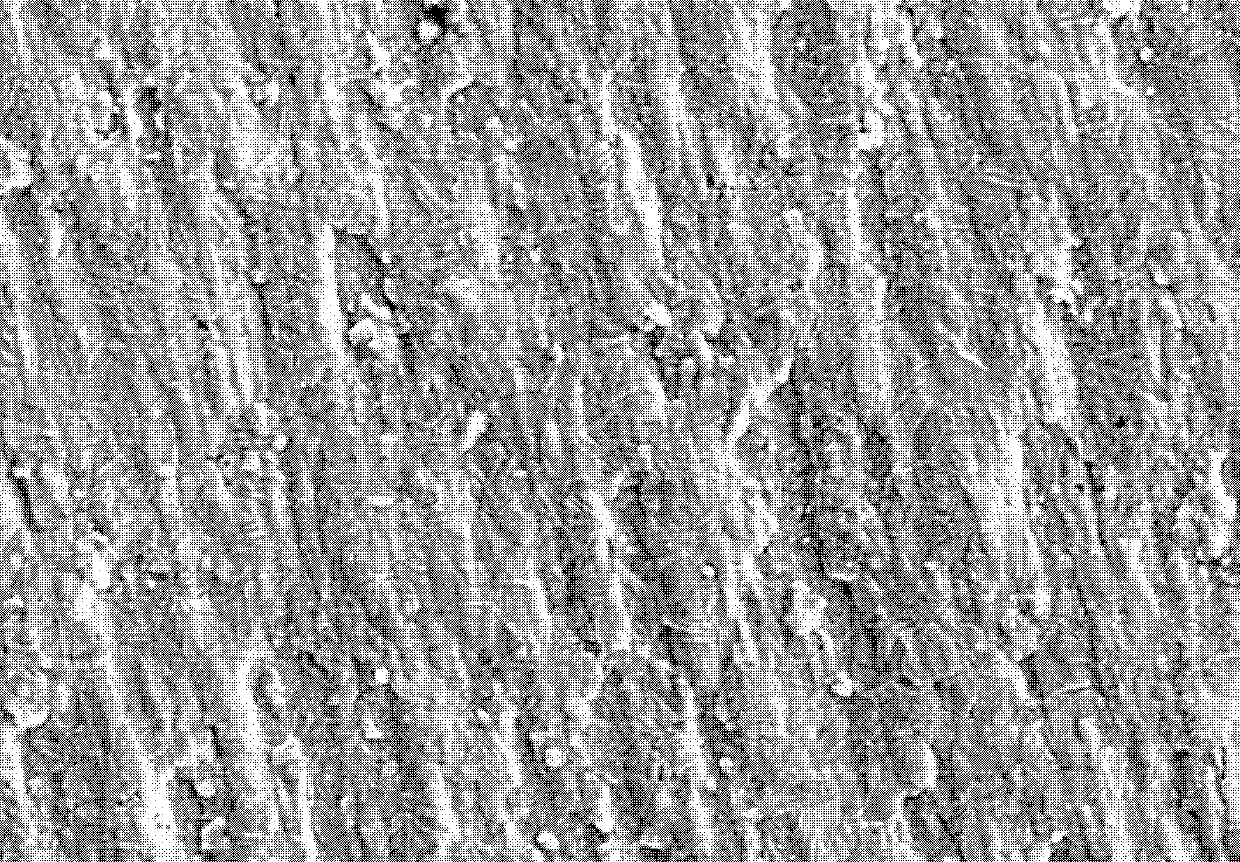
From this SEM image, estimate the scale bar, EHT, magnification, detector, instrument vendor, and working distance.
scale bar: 20000 nm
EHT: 15 kV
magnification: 2 K X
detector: SE(M)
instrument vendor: Hitachi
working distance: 12.6 mm